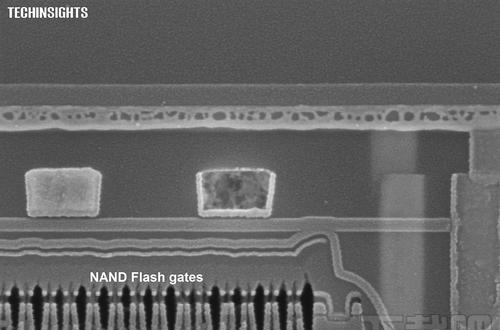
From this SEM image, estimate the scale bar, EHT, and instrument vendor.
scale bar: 200 nm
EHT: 5 kV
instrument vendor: Zeiss